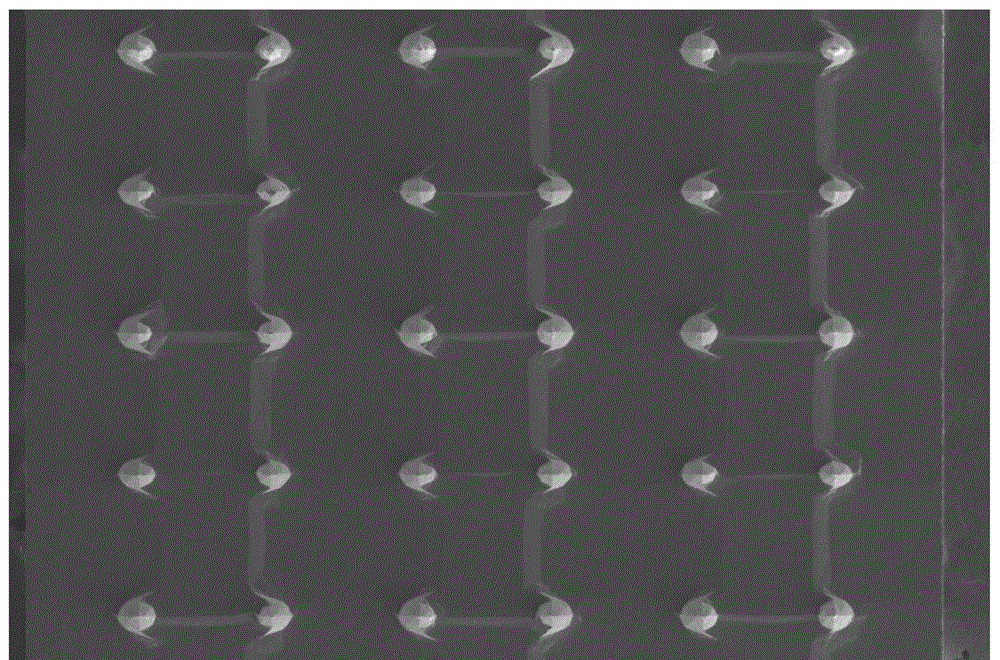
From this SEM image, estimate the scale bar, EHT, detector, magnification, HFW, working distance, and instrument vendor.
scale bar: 500000 nm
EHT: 5 kV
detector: ETD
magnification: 0.1 K X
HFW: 2070 µm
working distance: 5.4 mm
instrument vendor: FEI